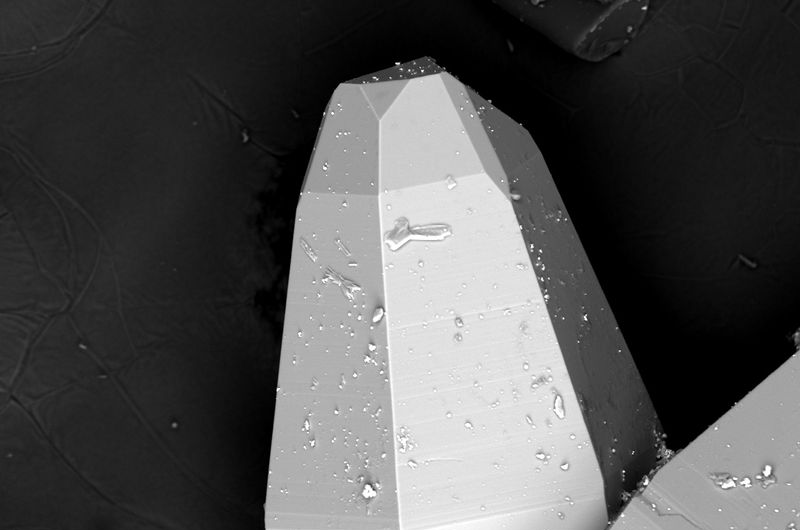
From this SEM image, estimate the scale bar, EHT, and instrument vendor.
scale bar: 100000 nm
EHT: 20 kV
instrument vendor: JEOL